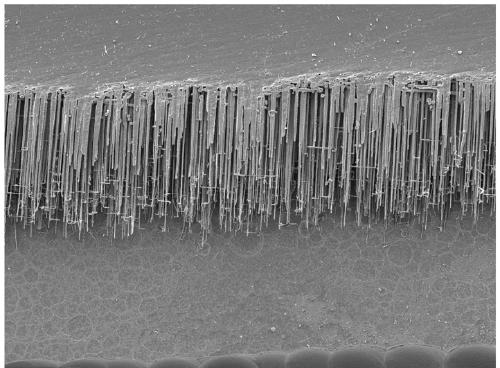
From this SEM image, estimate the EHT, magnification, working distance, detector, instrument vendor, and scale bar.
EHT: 5 kV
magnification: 0.6 K X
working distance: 10 mm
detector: LED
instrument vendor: JEOL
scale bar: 10000 nm